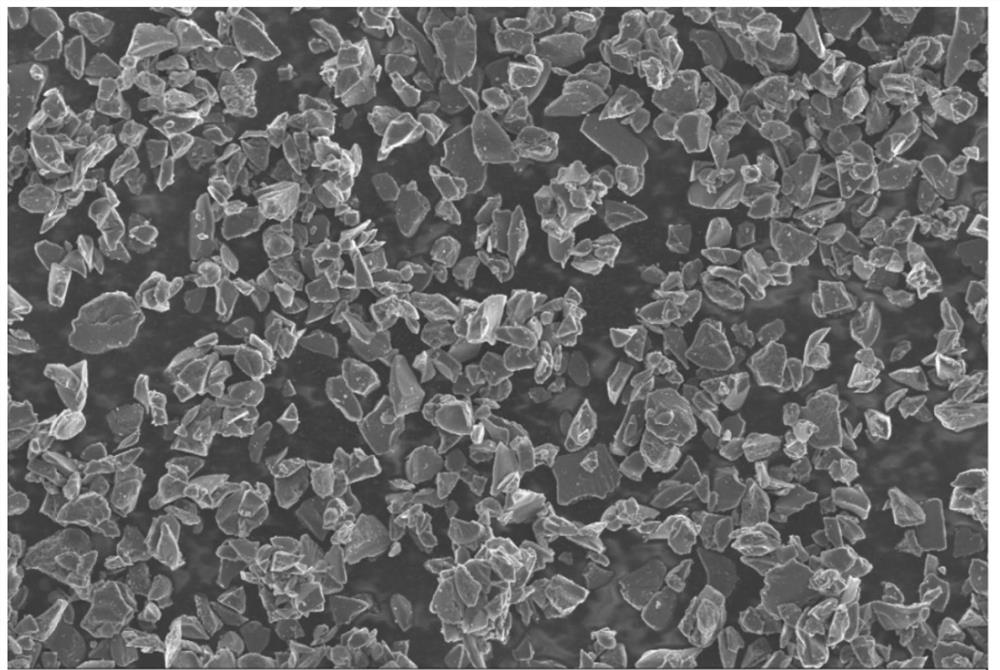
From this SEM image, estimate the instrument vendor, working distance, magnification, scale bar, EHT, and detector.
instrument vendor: Zeiss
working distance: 3.1 mm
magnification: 1 K X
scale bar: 10000 nm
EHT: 5 kV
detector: InLens